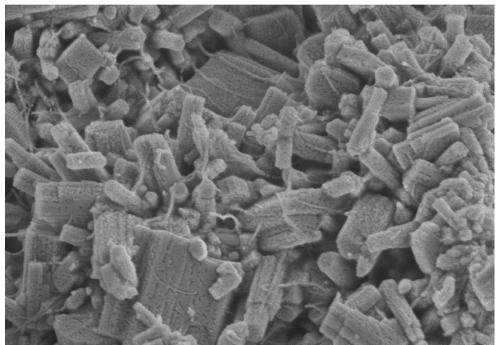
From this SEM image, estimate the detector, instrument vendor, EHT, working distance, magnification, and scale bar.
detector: SE(M)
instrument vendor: Hitachi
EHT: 3 kV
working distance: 10.5 mm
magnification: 50 K X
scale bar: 1000 nm